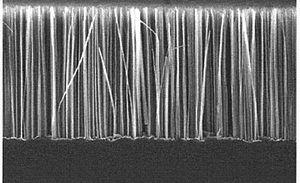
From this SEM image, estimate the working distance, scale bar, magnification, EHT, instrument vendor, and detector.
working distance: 5 mm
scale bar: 10000 nm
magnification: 5 K X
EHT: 5 kV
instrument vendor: Hitachi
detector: SE(M)